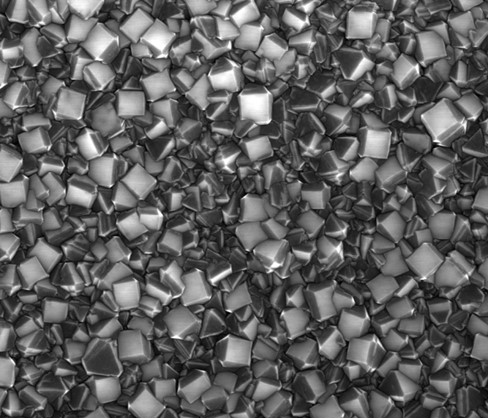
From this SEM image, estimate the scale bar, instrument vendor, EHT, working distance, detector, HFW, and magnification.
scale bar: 3000 nm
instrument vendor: FEI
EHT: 10 kV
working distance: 4.1 mm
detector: ETD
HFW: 8.54 µm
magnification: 14.996 K X